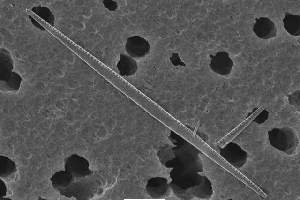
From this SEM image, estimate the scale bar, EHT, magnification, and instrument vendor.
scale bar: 5000 nm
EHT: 10 kV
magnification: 3.5 K X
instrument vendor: JEOL